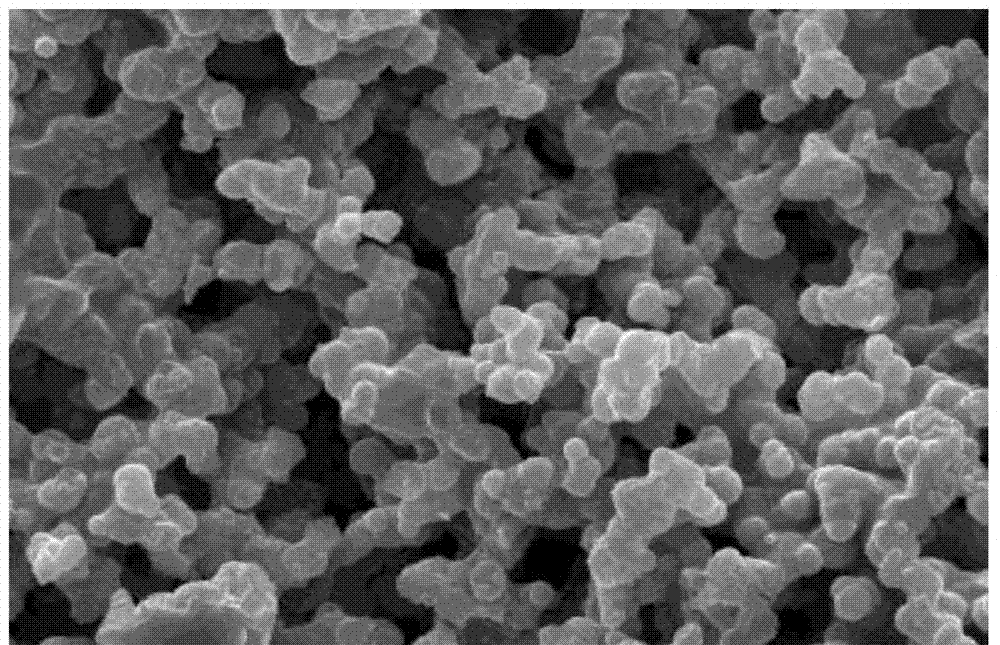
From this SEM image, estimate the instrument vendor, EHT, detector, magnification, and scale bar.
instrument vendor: JEOL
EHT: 20 kV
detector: SEI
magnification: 2 K X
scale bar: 10000 nm